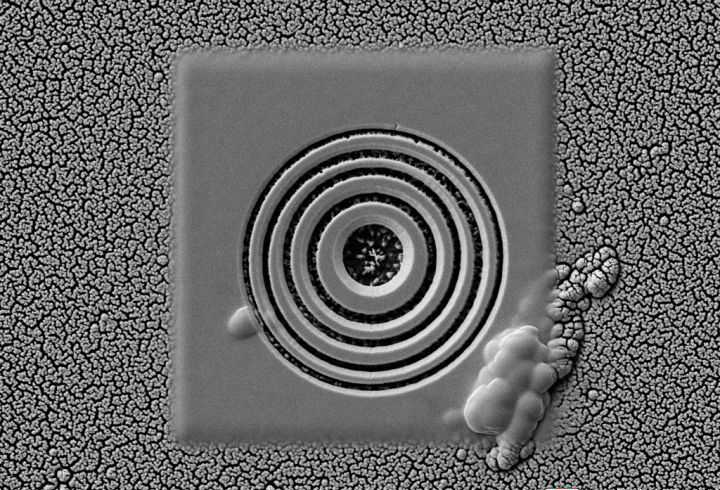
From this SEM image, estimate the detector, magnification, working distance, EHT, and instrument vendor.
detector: SE2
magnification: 5 K X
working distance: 8.3 mm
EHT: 5 kV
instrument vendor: Zeiss